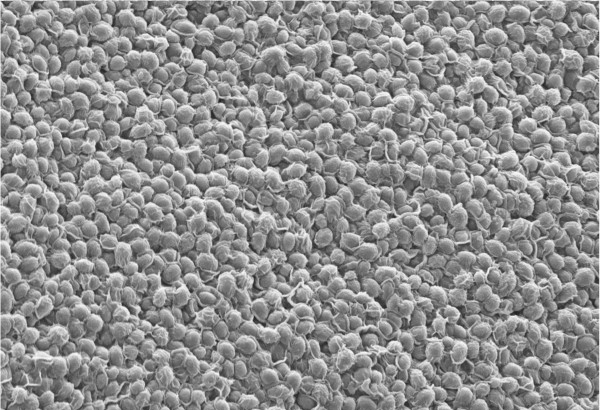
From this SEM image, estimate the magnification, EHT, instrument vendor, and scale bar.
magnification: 3 K X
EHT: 10 kV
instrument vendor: Zeiss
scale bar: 10000 nm